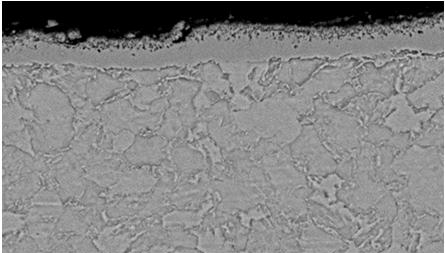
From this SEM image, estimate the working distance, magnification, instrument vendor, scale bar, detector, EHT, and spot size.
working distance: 10.9 mm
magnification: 1.5 K X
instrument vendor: FEI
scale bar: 20000 nm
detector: BSE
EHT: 20 kV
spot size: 3.5